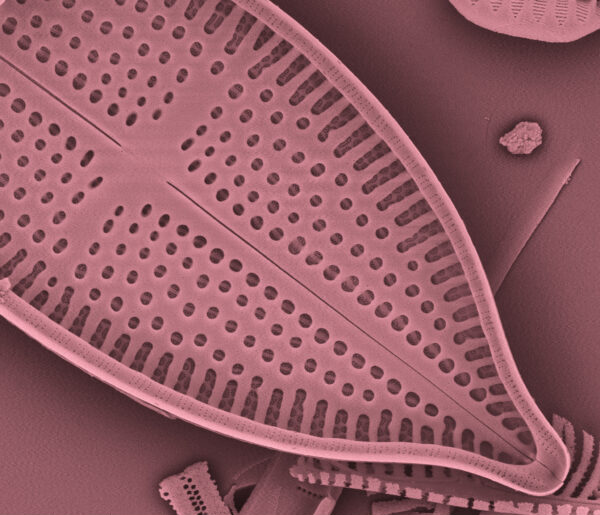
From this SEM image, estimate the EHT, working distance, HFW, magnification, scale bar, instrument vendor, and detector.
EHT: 5 kV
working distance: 9 mm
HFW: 25.1 µm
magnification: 5.948 K X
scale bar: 10000 nm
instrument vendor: FEI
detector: ETD